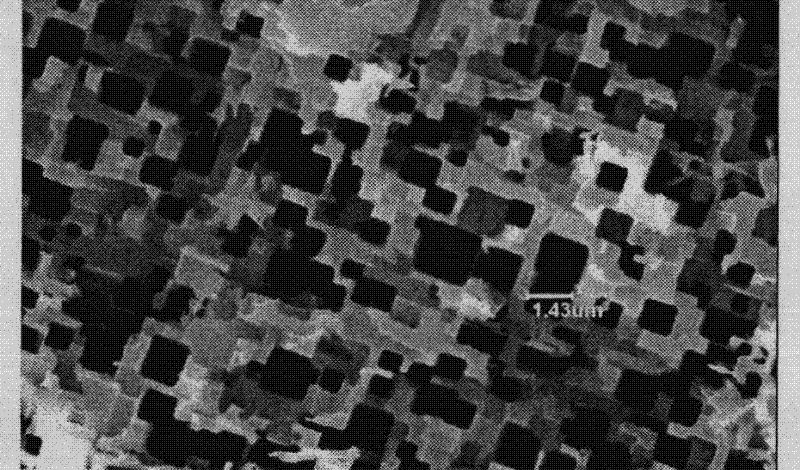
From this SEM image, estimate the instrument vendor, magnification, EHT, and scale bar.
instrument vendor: other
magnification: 5 K X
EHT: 20 kV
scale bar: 10000 nm